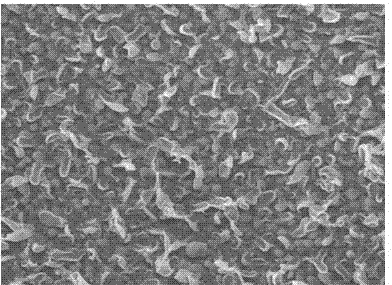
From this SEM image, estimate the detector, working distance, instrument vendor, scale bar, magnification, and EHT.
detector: LEI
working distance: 8.3 mm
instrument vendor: JEOL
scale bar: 1000 nm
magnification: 20 K X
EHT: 5 kV